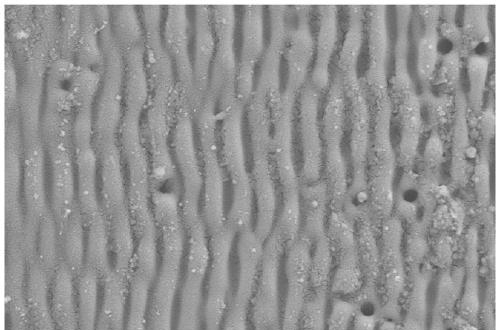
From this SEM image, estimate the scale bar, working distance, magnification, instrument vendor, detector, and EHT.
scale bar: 1000 nm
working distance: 3.6 mm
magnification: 20 K X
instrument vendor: Zeiss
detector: SE2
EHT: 5 kV